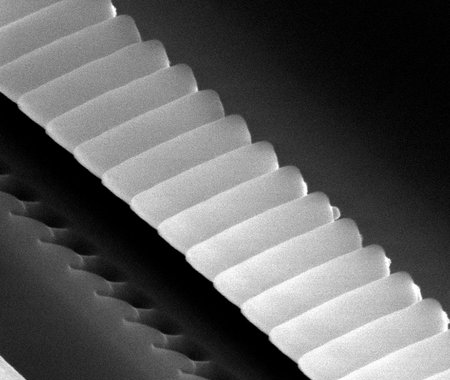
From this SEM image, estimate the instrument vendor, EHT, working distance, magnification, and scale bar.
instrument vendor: FEI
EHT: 20 kV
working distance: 4.9 mm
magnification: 50.008 K X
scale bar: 1000 nm